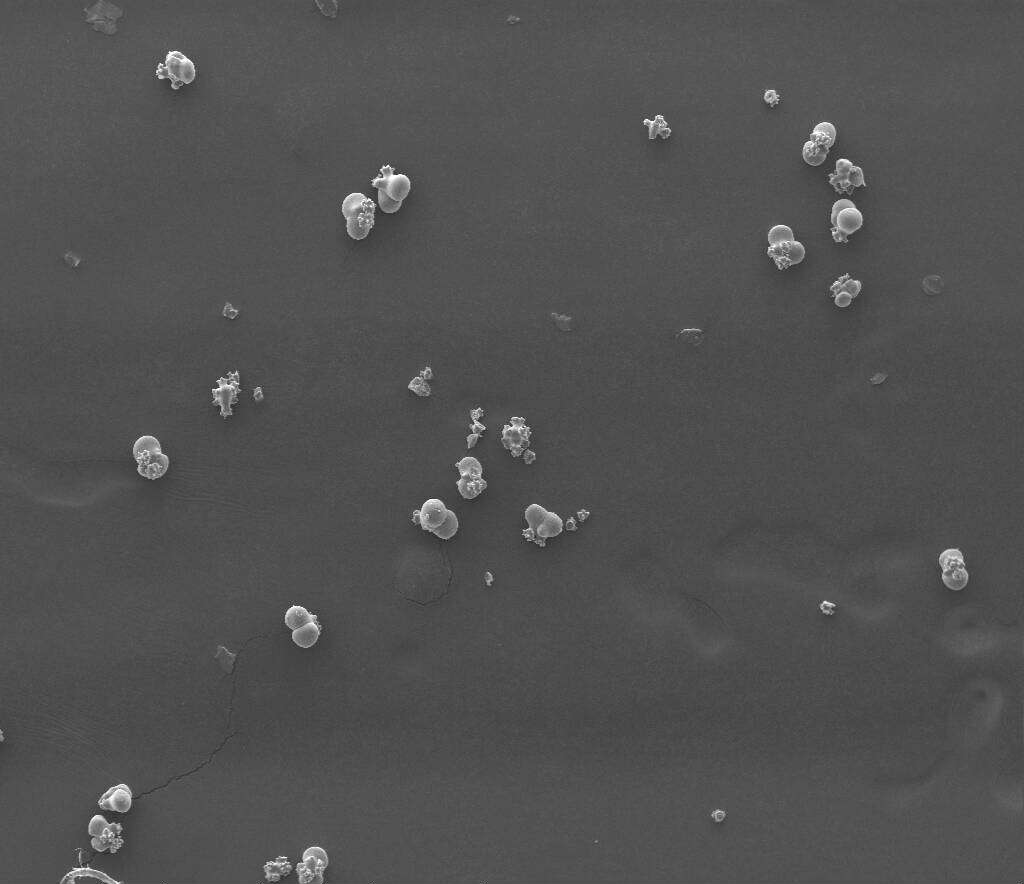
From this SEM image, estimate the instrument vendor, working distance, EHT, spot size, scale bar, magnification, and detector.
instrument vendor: FEI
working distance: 7.5 mm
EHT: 15 kV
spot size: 4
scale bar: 500000 nm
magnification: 0.2 K X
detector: SE